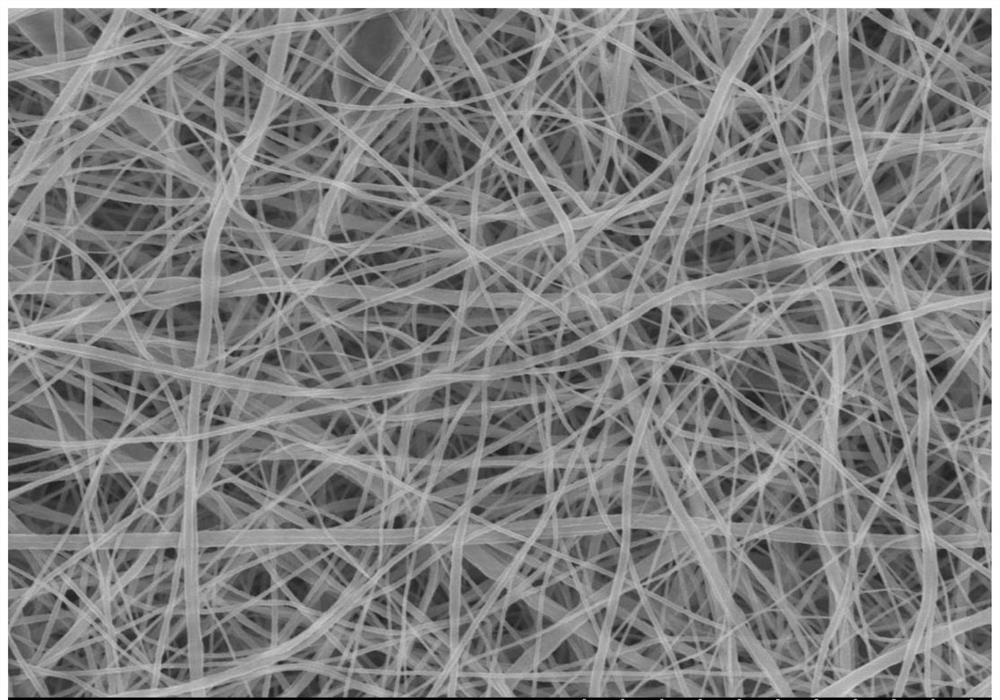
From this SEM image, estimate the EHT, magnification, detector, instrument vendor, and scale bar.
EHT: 8 kV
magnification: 5 K X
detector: SE(U)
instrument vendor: Hitachi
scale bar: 10000 nm